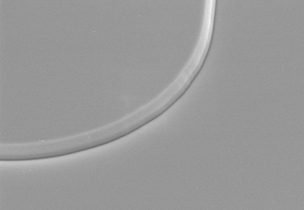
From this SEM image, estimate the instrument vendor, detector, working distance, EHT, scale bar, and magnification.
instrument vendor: Hitachi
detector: SE(M)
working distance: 7.4 mm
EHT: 15 kV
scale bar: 10000 nm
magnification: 4 K X